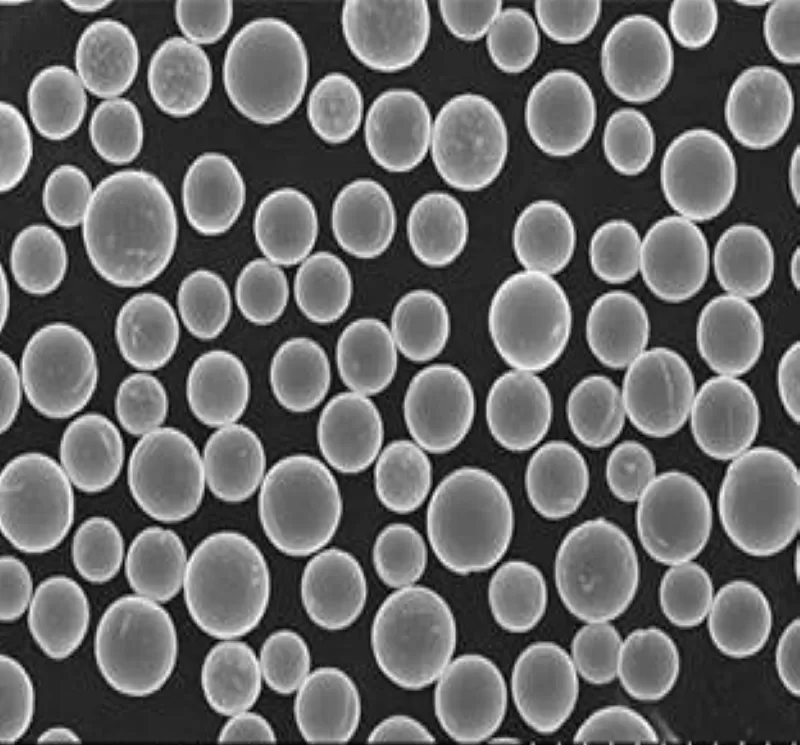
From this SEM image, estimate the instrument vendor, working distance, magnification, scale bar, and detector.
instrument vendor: Hitachi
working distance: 10.6 mm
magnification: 1 K X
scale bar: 100000 nm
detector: SE(U)